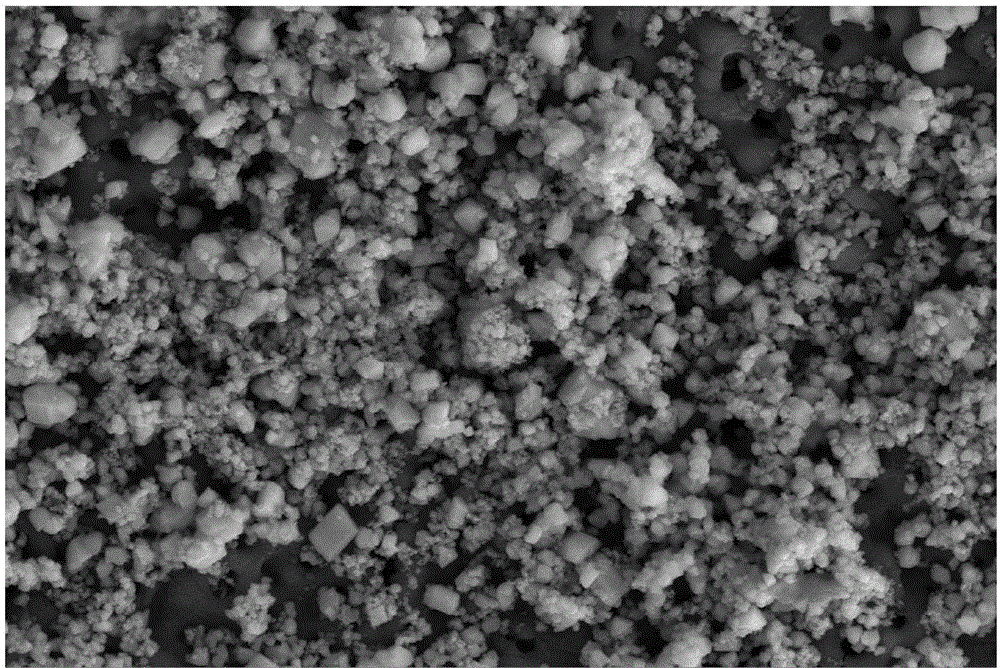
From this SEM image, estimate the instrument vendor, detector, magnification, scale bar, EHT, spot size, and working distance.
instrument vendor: FEI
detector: ETD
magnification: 20 K X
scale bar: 5000 nm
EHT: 15 kV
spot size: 3.5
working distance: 7.2 mm